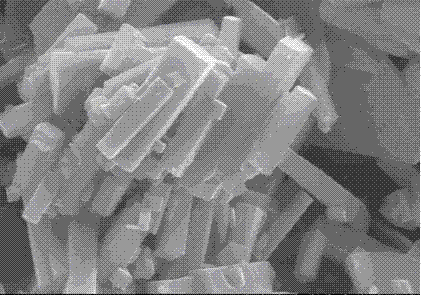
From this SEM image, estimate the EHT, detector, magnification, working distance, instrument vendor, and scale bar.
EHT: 15 kV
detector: SE(M)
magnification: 10 K X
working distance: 16.8 mm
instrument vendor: Hitachi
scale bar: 5000 nm